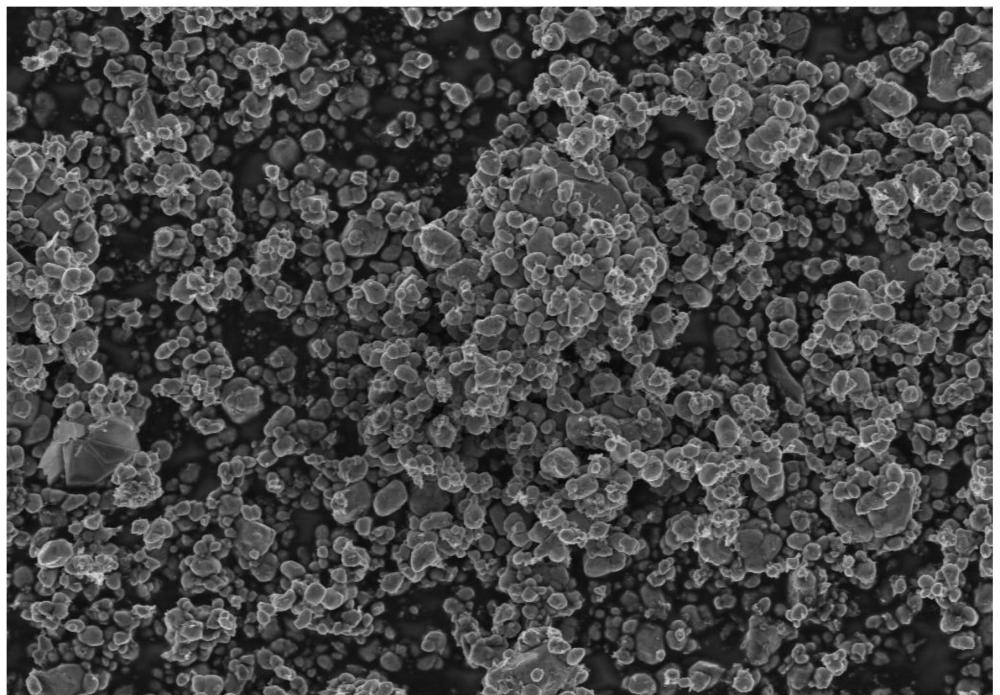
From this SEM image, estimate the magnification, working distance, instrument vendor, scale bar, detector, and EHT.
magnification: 5 K X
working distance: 4.6 mm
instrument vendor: Zeiss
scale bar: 2000 nm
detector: InLens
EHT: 3 kV